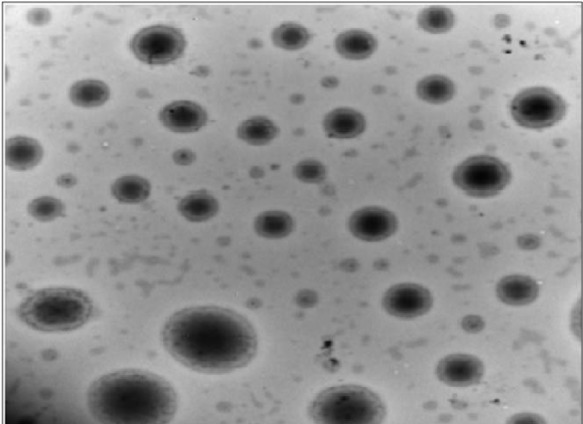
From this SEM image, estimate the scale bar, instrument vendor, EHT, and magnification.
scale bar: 500 nm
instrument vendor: FEI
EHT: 80 kV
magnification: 10 K X